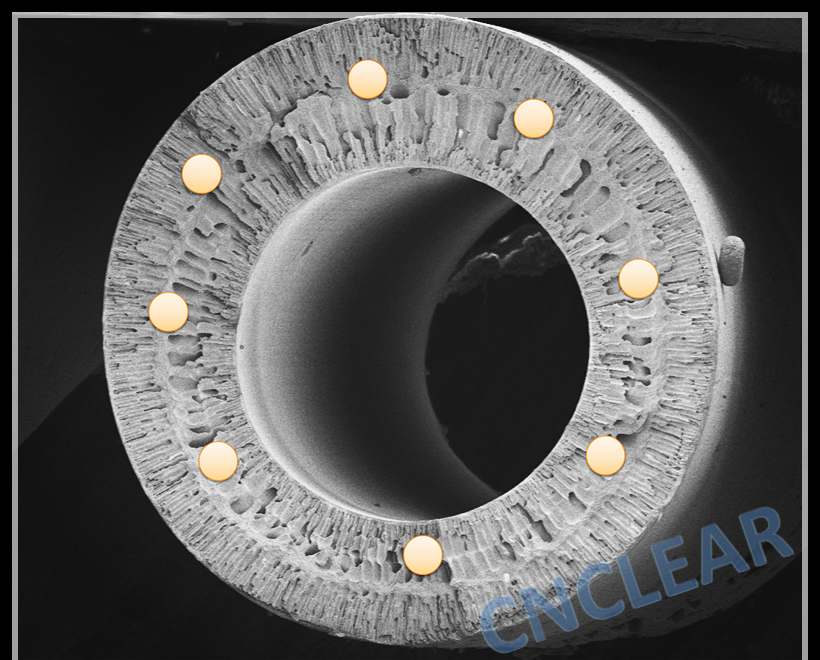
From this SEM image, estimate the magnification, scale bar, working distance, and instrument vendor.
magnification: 0.07 K X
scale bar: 500000 nm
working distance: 6.9 mm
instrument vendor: Hitachi